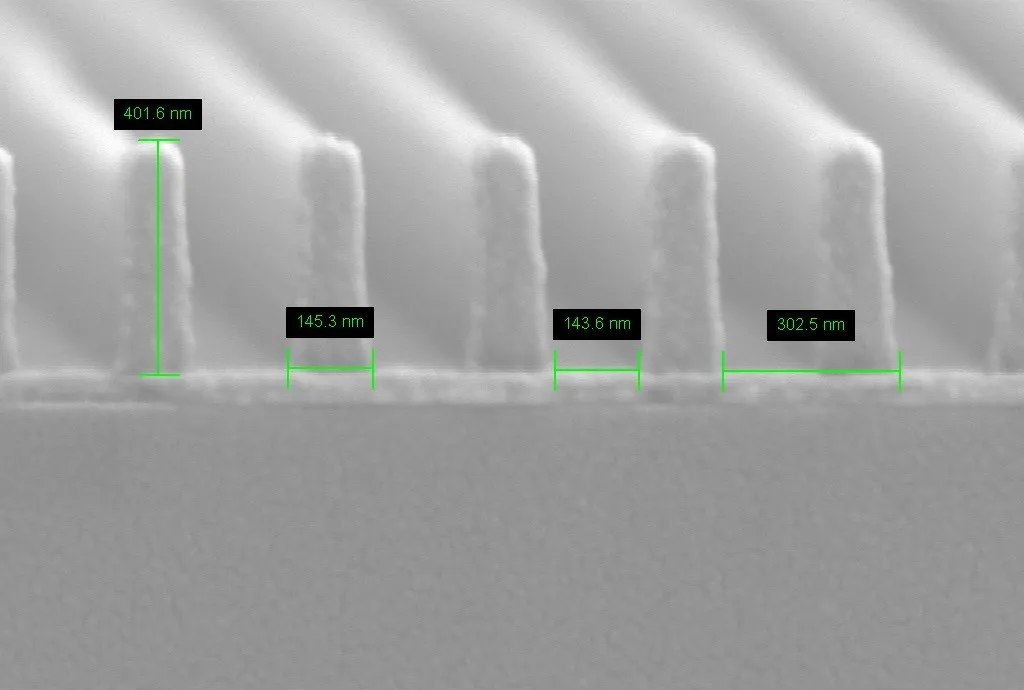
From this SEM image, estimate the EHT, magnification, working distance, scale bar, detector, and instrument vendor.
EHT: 3 kV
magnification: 160 K X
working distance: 7.7 mm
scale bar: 100 nm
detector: SE2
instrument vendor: Zeiss